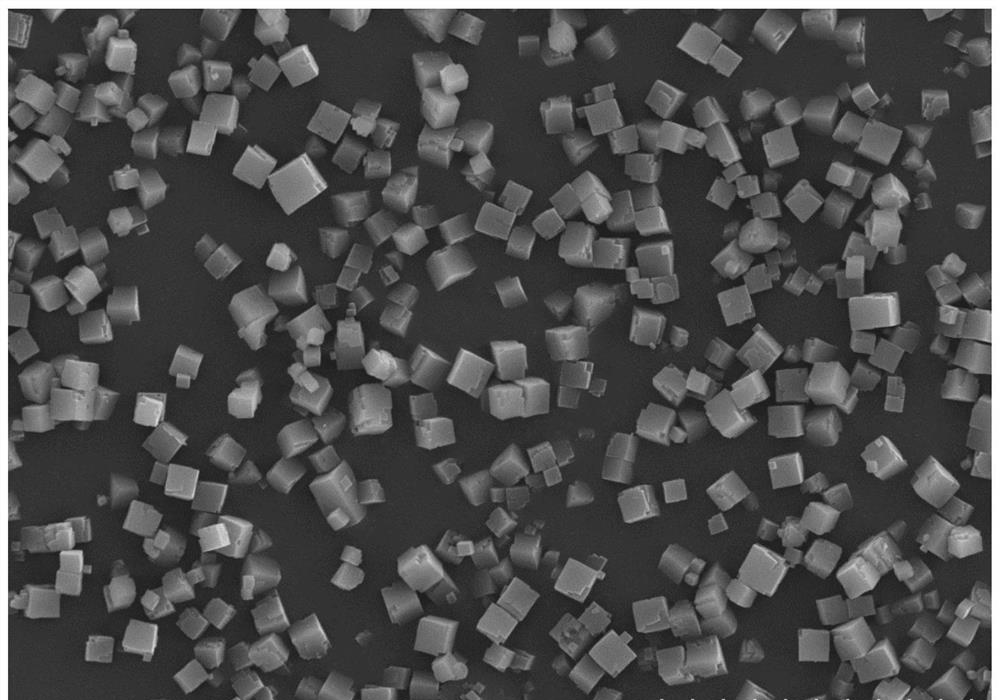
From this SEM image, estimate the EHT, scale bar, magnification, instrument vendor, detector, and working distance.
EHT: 15 kV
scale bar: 20000 nm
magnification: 2 K X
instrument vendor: Hitachi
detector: SE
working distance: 6.7 mm